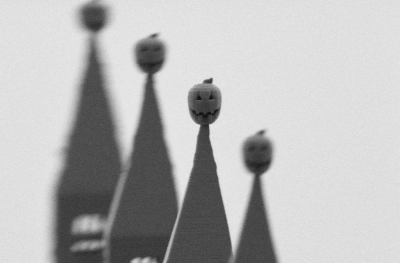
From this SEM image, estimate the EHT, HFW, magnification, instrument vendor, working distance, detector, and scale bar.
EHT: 1 kV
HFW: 149.2 µm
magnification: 1.7 K X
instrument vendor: Zeiss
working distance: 5.3 mm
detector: SE2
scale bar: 10000 nm